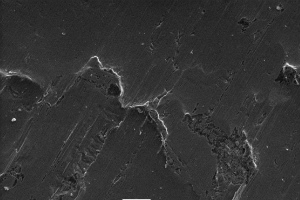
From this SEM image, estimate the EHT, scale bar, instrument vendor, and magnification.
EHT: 15 kV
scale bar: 20000 nm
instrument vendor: JEOL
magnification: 0.5 K X